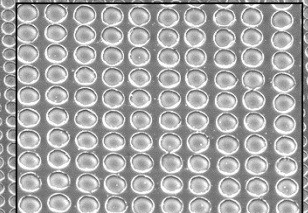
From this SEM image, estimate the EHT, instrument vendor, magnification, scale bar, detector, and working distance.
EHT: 10 kV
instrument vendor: JEOL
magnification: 2 K X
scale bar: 10000 nm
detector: SEI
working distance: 9.4 mm